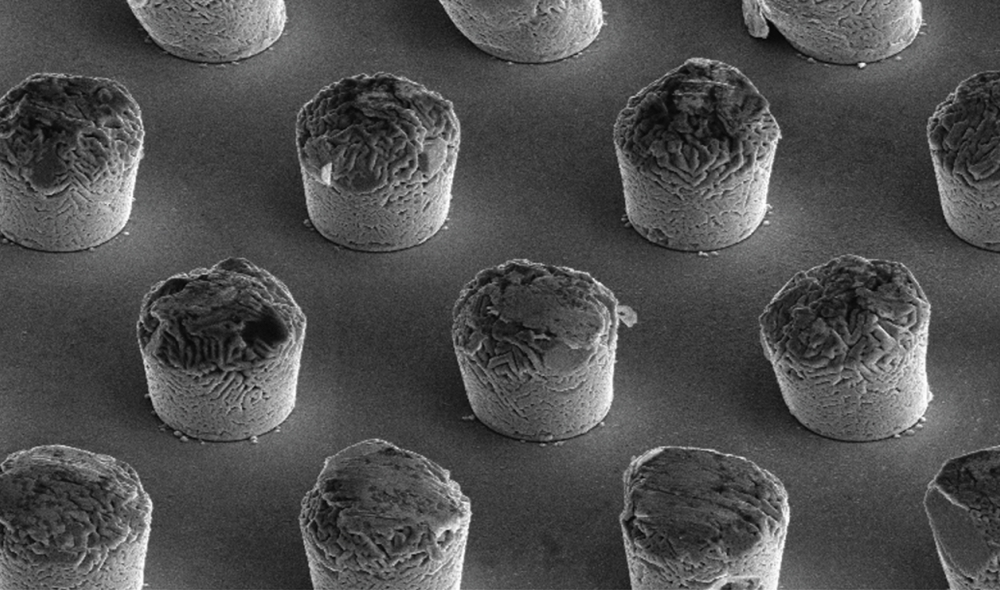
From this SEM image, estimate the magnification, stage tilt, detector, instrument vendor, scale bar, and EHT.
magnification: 2.5 K X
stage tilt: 52°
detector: ETD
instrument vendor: FEI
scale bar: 40000 nm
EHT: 10 kV